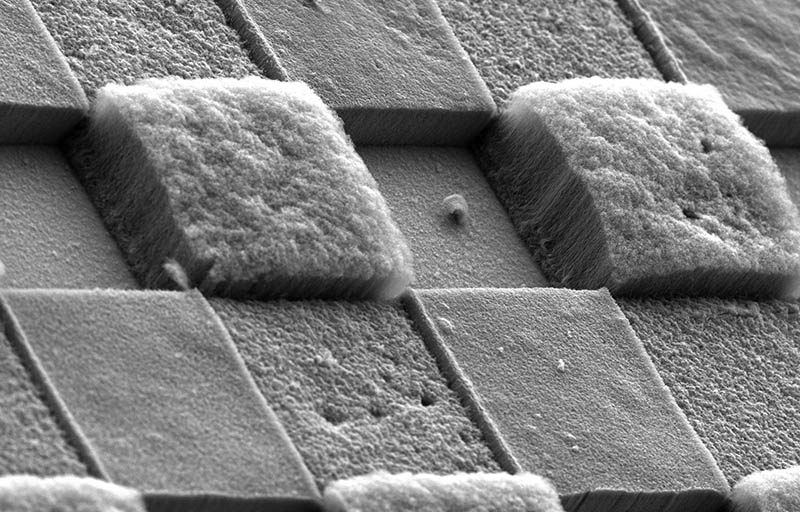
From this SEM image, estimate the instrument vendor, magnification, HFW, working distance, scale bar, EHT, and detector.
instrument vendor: FEI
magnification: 0.344 K X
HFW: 744 µm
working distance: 7.6 mm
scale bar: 400000 nm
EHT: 5 kV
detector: ETD